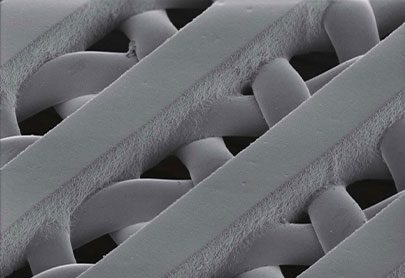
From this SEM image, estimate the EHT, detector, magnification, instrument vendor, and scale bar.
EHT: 1 kV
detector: SE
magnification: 0.35 K X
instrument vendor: Hitachi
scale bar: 100000 nm